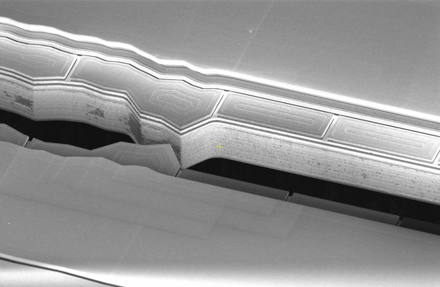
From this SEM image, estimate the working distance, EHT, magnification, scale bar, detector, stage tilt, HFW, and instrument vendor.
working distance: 4.1 mm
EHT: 5 kV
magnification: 1.5 K X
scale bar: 100000 nm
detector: TLD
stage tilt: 52°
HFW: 276 µm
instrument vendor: FEI